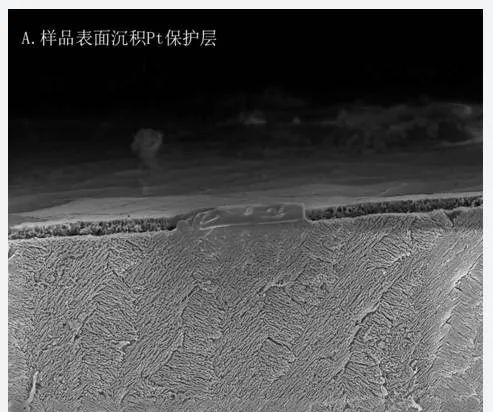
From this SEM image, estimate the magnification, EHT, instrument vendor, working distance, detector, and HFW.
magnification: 7 K X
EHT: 5 kV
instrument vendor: FEI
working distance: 4 mm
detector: ETD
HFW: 36.6 µm